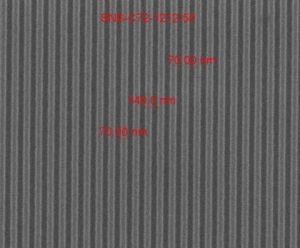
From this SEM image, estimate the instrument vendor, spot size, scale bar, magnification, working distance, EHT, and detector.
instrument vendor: FEI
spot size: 1.5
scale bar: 1000 nm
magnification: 75 K X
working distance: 10.5 mm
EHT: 15 kV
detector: ETD